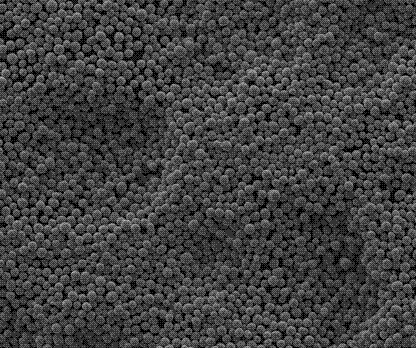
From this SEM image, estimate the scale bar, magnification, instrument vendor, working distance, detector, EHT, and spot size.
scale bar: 200000 nm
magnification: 0.4 K X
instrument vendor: FEI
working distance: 9.6 mm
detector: SE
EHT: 25 kV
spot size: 1.5